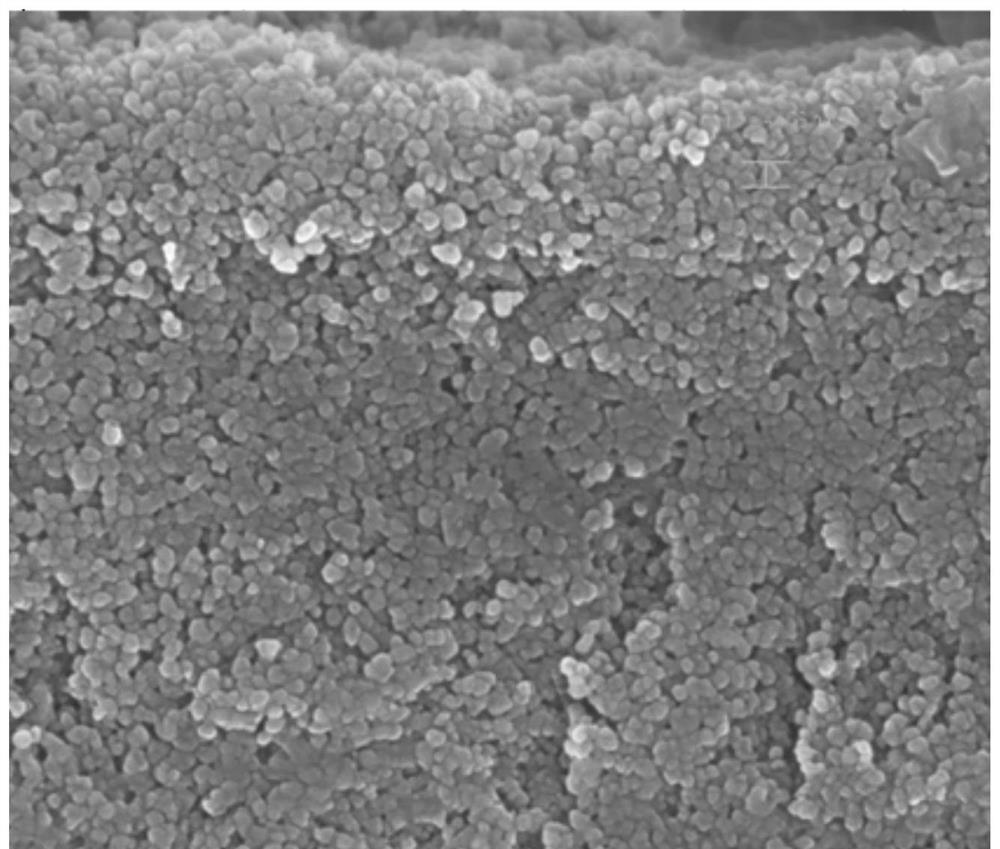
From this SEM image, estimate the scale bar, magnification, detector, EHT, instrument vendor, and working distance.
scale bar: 100 nm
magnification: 50 K X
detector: InLens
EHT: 5 kV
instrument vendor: Zeiss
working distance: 4.1 mm